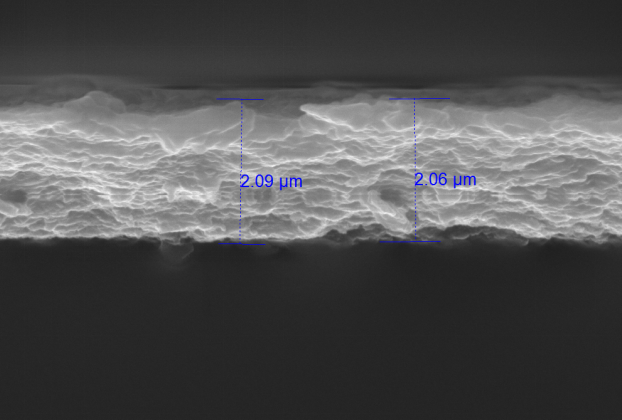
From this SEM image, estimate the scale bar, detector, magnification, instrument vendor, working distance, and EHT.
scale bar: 2000 nm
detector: SE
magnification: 30 K X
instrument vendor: Tescan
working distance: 7.99 mm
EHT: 15 kV